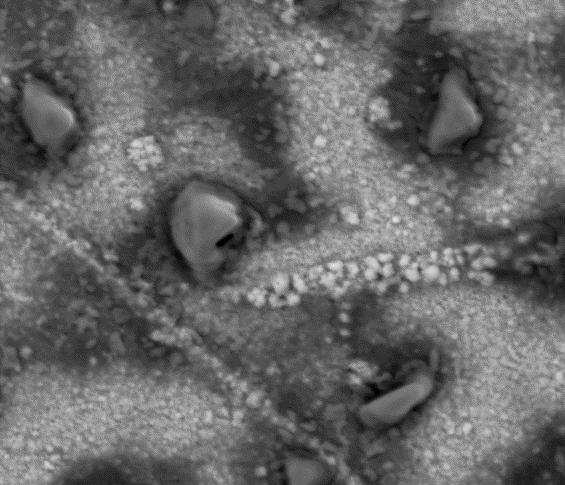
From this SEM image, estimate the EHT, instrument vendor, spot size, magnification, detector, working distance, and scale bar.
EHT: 15 kV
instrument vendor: FEI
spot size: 4.5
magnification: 3 K X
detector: Mix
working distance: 10.6 mm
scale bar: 20000 nm